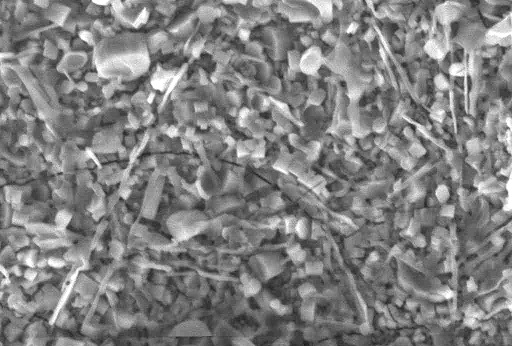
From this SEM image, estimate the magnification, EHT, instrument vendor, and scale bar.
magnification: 2 K X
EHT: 20 kV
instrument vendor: other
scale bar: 10000 nm